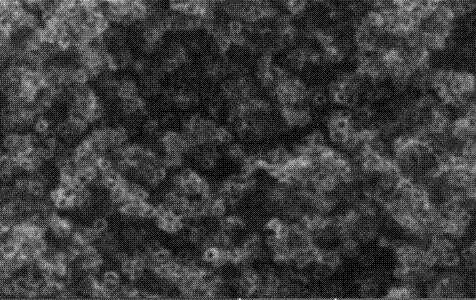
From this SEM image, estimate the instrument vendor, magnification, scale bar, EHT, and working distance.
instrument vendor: Tescan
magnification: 30 K X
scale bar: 1000 nm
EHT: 20 kV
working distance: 10.02 mm